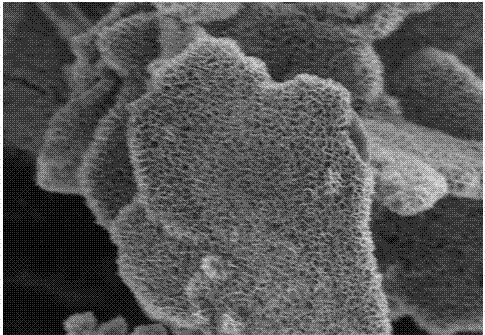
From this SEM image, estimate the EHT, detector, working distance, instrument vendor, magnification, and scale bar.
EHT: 3 kV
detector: SE(UL)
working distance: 11.4 mm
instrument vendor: Hitachi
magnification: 15 K X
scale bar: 3000 nm